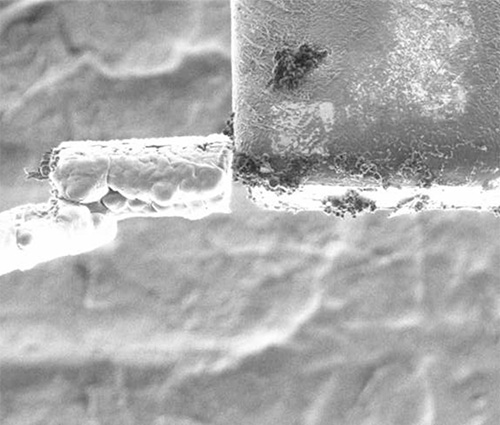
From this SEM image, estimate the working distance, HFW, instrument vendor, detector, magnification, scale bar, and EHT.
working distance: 18 mm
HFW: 60.8 µm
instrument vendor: FEI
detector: SED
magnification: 5 K X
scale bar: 10000 nm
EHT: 30 kV